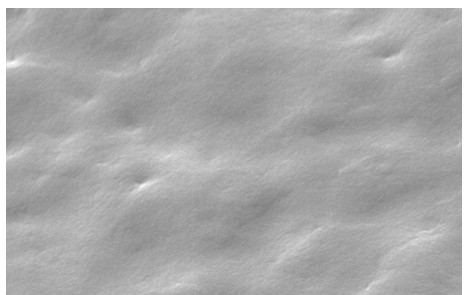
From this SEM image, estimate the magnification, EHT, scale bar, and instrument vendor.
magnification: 2 K X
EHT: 20 kV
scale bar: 10000 nm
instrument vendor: other